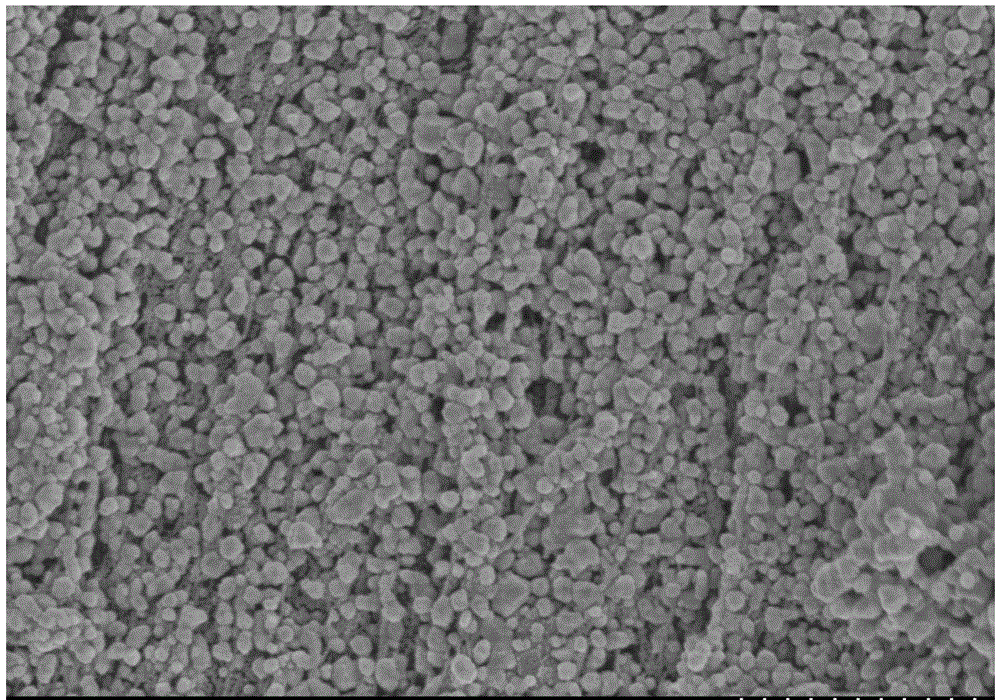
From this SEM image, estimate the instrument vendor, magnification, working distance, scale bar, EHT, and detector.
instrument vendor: Hitachi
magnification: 30 K X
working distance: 8.6 mm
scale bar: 1000 nm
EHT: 3 kV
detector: SE(M)